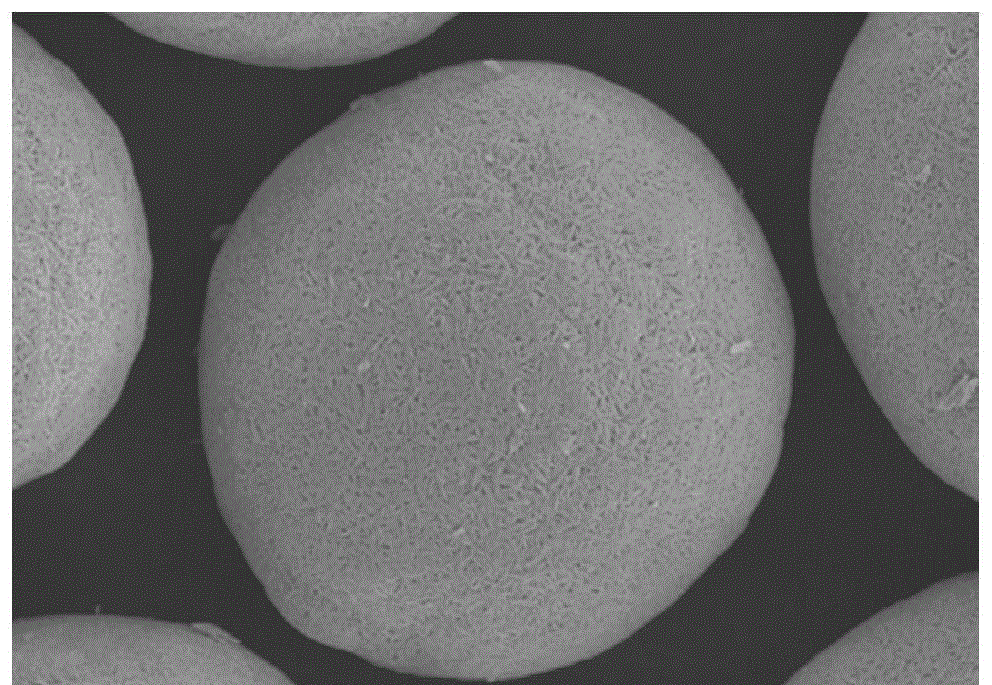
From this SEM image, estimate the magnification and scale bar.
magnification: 5 K X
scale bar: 5000 nm